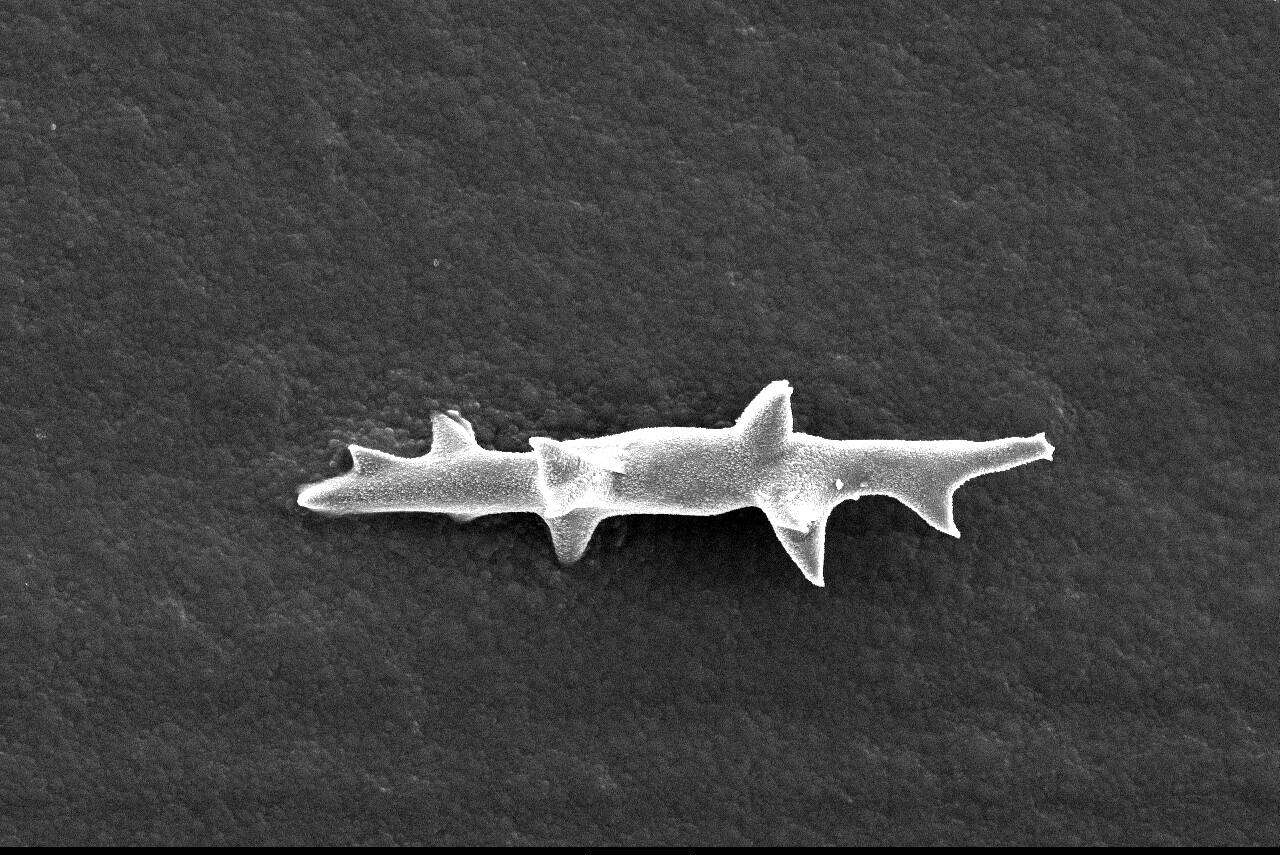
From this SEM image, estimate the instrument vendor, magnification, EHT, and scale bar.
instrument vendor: other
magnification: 1 K X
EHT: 10 kV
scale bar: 10000 nm